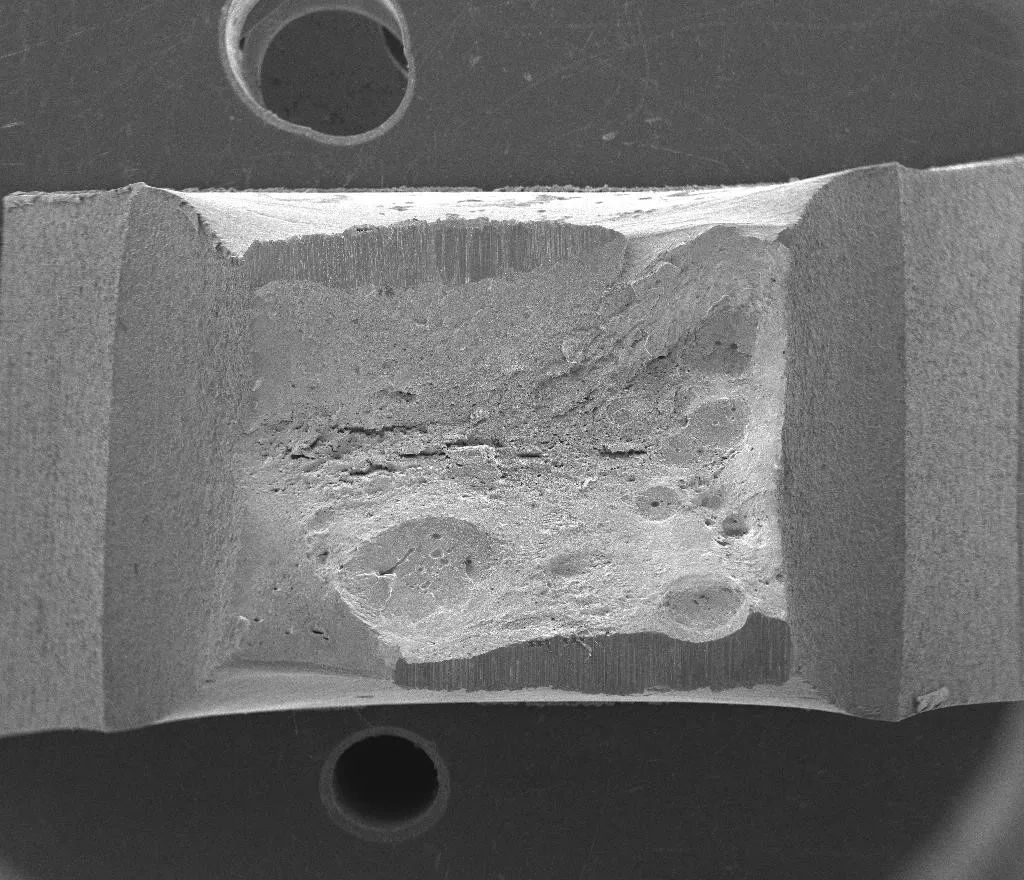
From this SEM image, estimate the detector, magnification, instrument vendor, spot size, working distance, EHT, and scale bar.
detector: ETD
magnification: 0.008 K X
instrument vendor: FEI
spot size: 4.5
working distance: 55.5 mm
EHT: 20 kV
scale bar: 5000000 nm